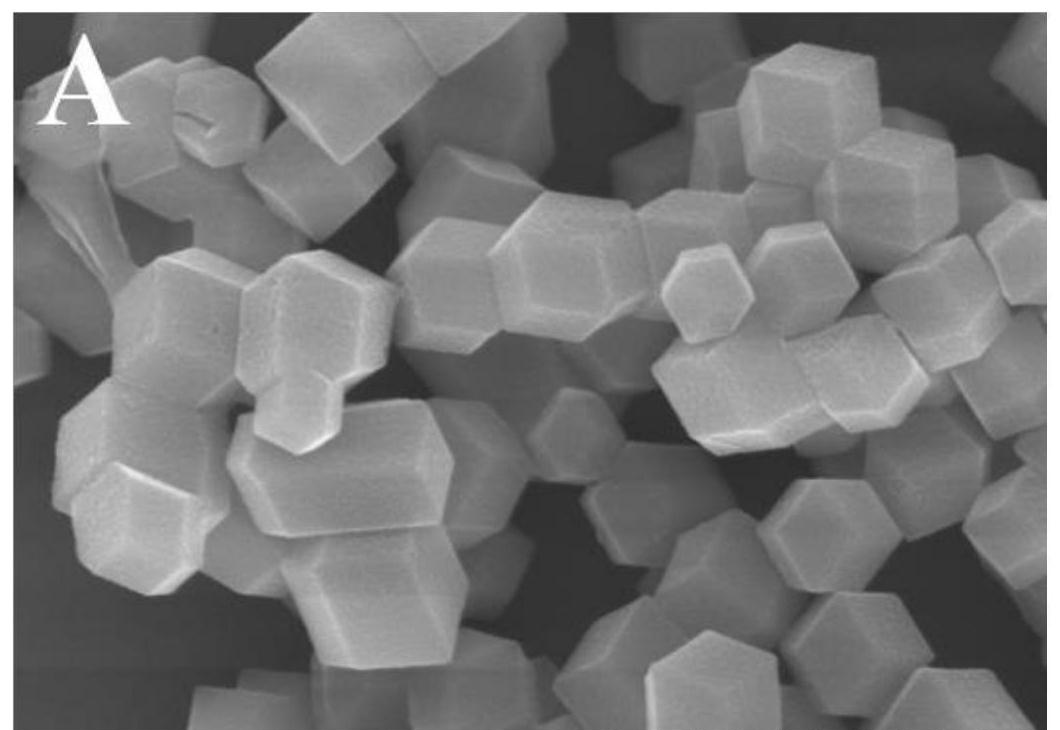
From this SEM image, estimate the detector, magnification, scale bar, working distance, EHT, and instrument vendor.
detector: SE(UL)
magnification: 50 K X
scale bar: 1000 nm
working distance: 8.7 mm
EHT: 5 kV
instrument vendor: Hitachi